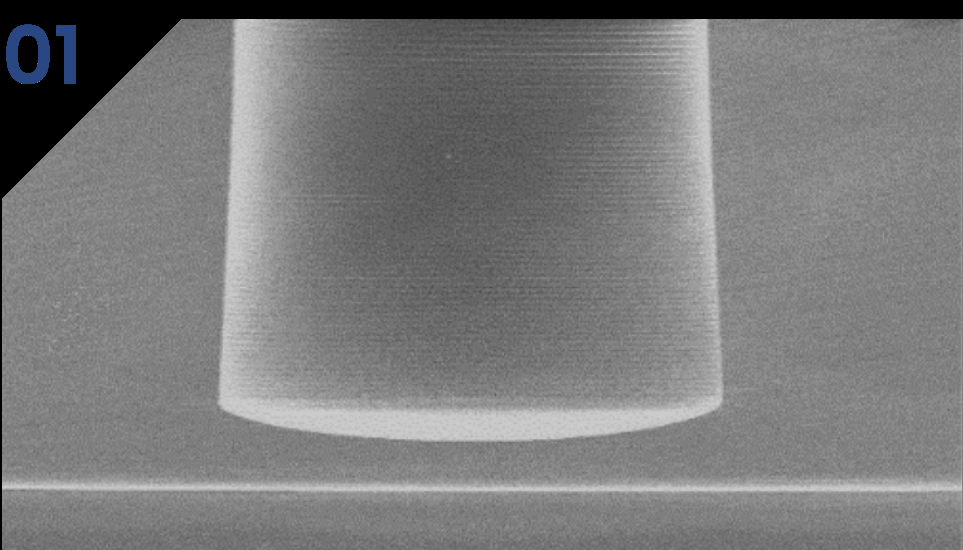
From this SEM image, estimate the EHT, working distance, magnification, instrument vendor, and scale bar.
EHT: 5 kV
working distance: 12 mm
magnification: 0.1 K X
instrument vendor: Hitachi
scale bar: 500000 nm